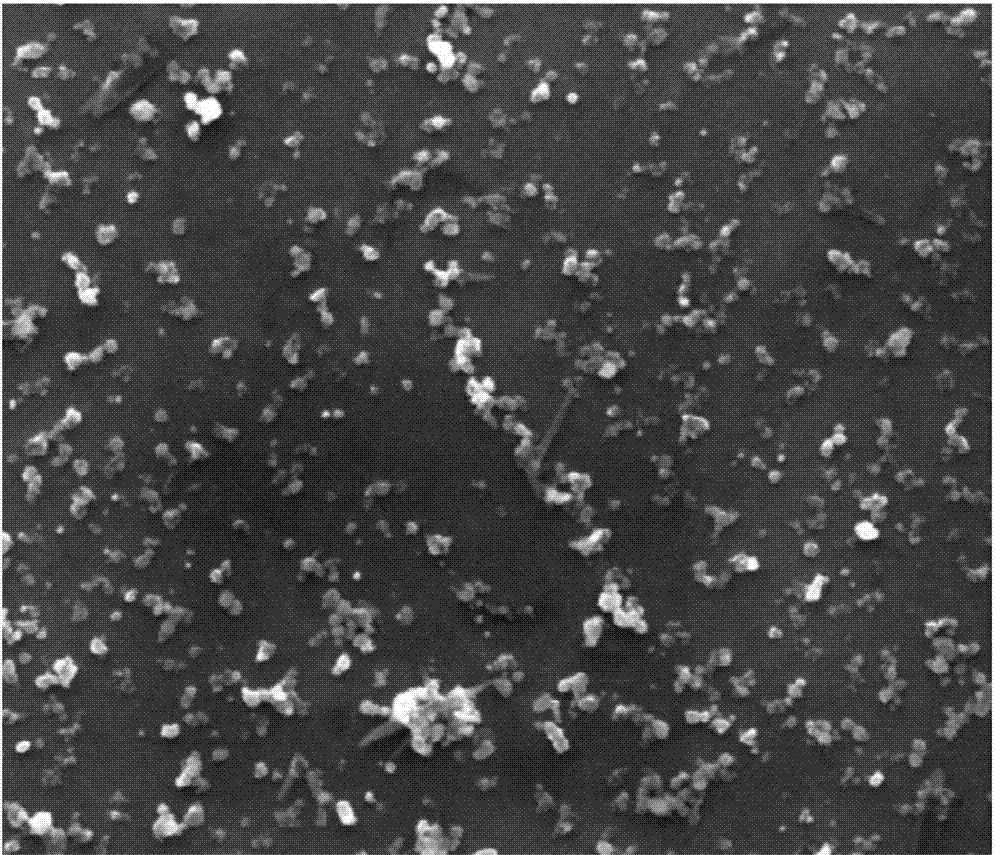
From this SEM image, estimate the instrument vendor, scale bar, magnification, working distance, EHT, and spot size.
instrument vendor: FEI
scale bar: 2000 nm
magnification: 50 K X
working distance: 9.8 mm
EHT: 20 kV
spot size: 3